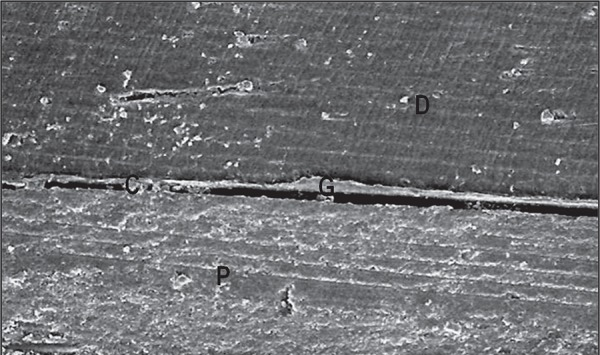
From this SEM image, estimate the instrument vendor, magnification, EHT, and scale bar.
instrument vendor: JEOL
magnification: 0.2 K X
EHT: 15 kV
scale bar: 100000 nm